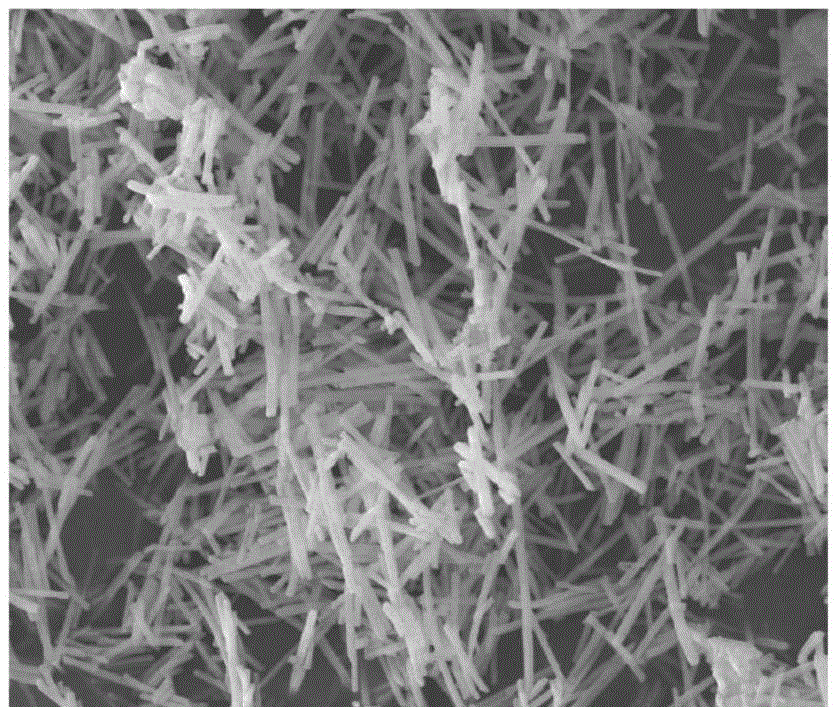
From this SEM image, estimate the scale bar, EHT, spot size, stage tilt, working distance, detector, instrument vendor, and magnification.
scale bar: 10000 nm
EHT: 10 kV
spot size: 3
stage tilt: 0°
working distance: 8.7 mm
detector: ETD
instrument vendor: FEI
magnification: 10 K X